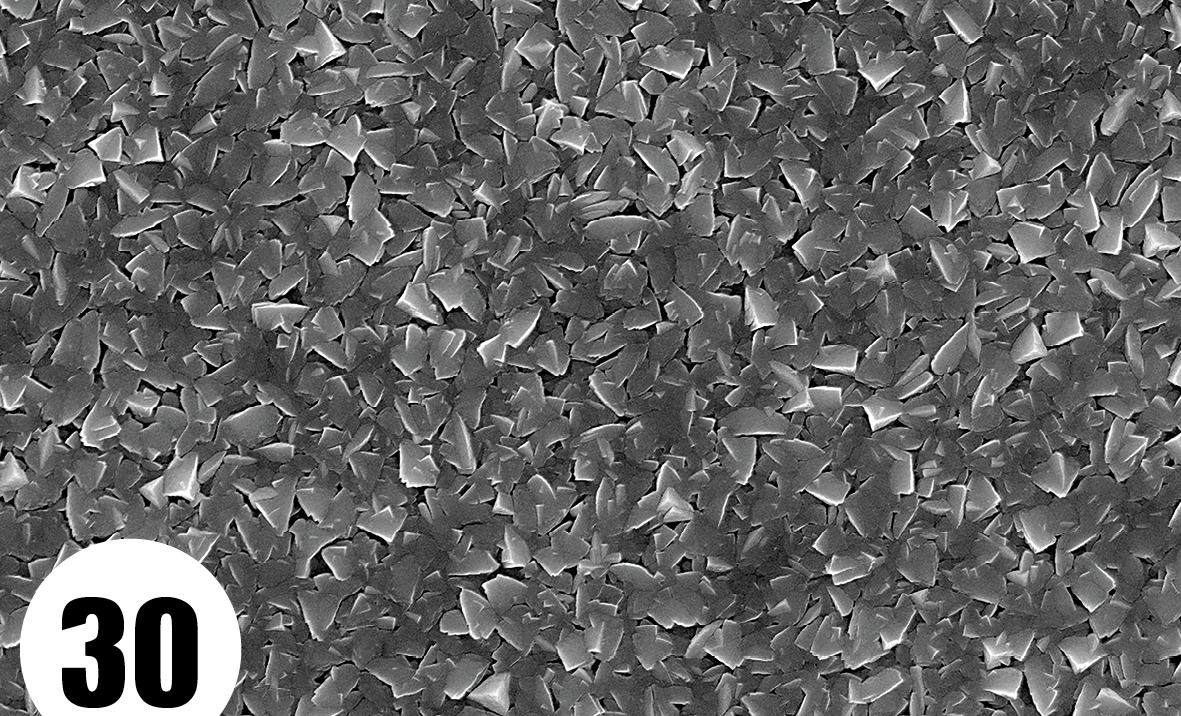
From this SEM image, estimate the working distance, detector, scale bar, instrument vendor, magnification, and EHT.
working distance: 4.5 mm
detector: SEI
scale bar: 1000 nm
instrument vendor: JEOL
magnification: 10 K X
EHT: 15 kV